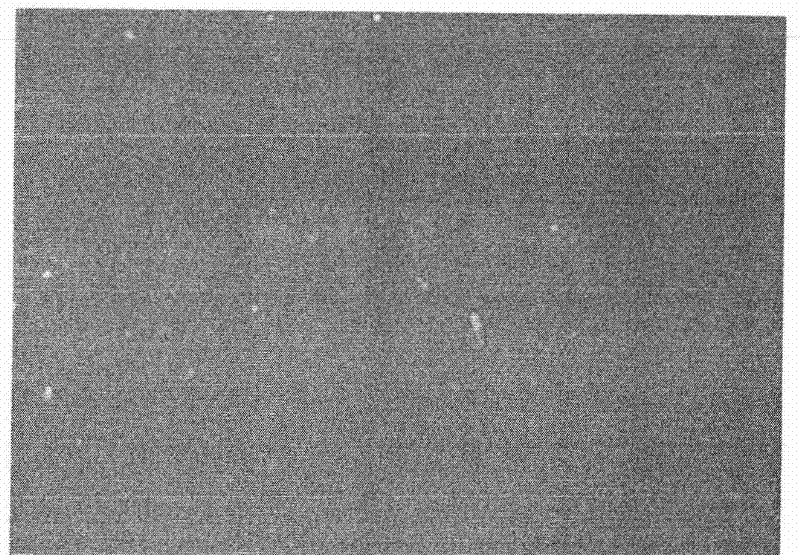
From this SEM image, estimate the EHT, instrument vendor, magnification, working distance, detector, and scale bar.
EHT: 10 kV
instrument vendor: Hitachi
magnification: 1 K X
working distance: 8.4 mm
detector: SE(M)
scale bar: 50000 nm